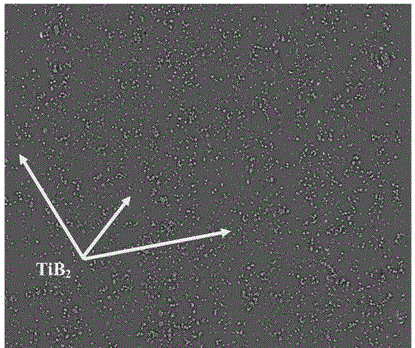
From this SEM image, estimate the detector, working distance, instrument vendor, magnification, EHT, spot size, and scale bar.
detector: vCD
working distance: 11.8 mm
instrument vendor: FEI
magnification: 1 K X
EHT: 20 kV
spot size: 5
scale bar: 100000 nm